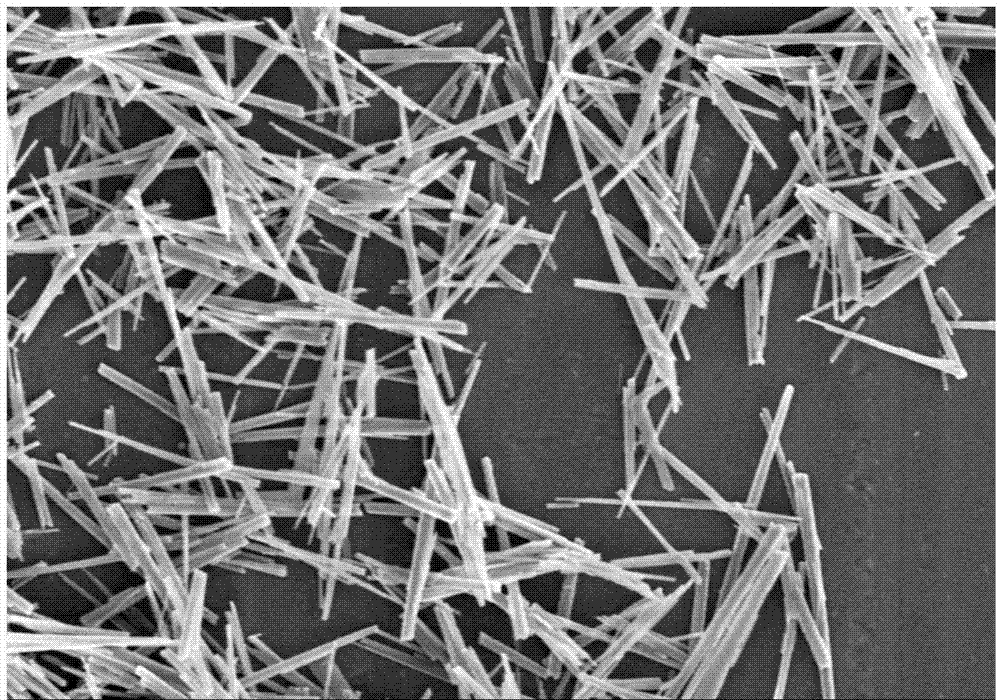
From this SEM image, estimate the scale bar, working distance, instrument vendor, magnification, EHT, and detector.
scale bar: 50000 nm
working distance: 9.6 mm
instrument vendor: Hitachi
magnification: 1 K X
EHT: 10 kV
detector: SE(M)